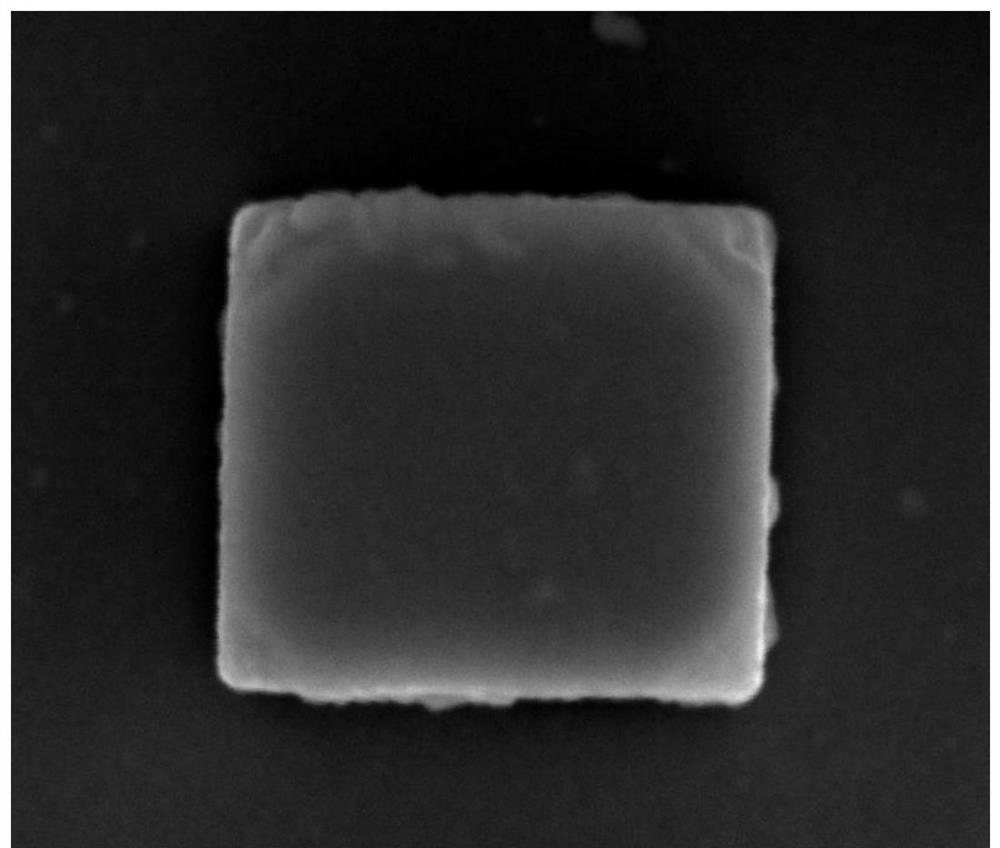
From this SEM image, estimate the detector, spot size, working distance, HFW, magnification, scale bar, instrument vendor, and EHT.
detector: ETD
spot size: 2.5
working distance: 4.3 mm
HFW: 1.49 µm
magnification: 100 K X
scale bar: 500 nm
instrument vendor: FEI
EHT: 8 kV